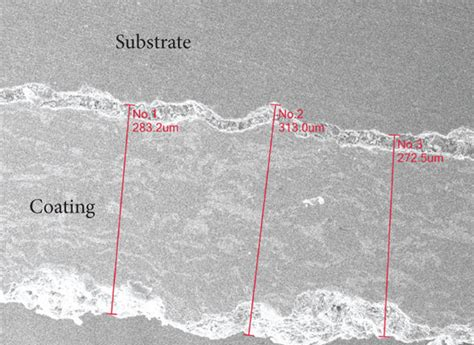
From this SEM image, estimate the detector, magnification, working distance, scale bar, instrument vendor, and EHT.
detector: SED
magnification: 0.2 K X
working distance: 9.8 mm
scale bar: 100000 nm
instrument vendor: JEOL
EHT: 20 kV